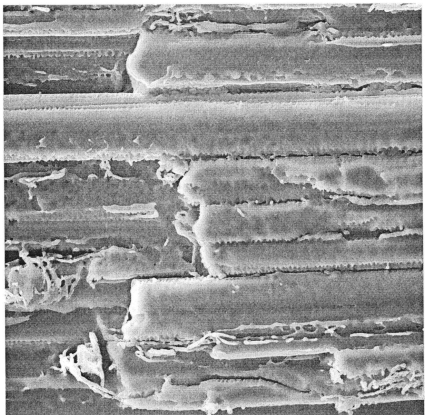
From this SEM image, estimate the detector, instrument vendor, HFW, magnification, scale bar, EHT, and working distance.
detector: SE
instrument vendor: Tescan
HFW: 40 µm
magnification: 7.22 K X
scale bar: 10000 nm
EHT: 10 kV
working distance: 8.65 mm